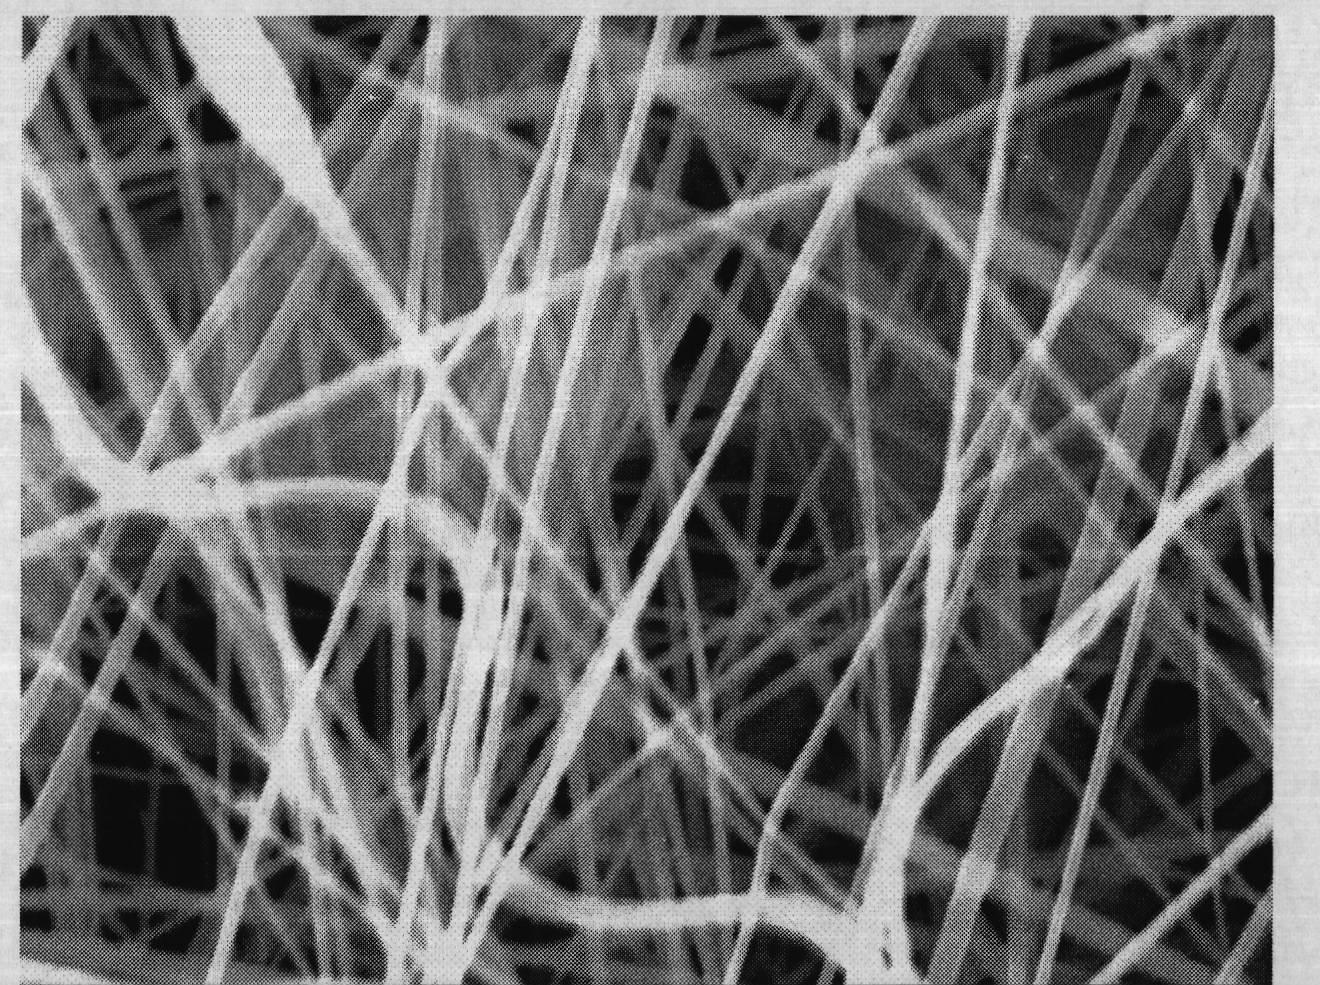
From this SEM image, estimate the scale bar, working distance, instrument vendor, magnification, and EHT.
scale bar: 1000 nm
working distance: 9 mm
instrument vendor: Zeiss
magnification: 15 K X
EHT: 15 kV